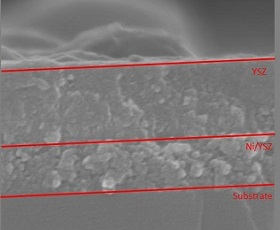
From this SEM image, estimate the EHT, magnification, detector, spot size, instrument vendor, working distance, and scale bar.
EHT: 20 kV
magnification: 300 K X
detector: TLD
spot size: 4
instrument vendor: FEI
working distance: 5.5 mm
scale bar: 500 nm